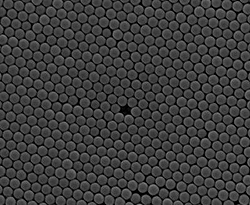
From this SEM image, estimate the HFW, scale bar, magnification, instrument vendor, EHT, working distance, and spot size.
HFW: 5.12 µm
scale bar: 2000 nm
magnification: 25 K X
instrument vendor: FEI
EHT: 10 kV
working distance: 9.9 mm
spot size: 3